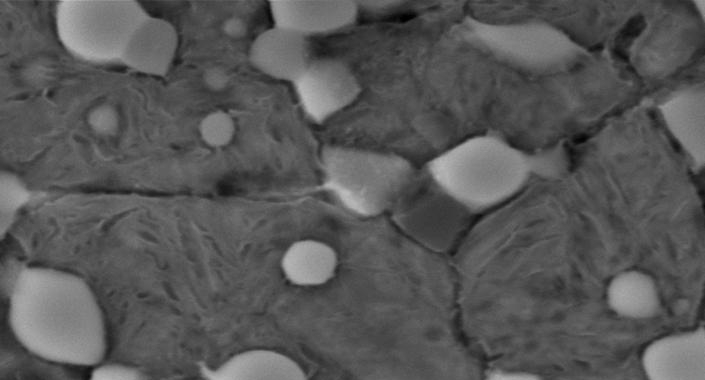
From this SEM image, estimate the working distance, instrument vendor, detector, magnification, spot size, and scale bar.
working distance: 11.8 mm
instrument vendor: FEI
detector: SE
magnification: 10 K X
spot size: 4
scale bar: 2000 nm